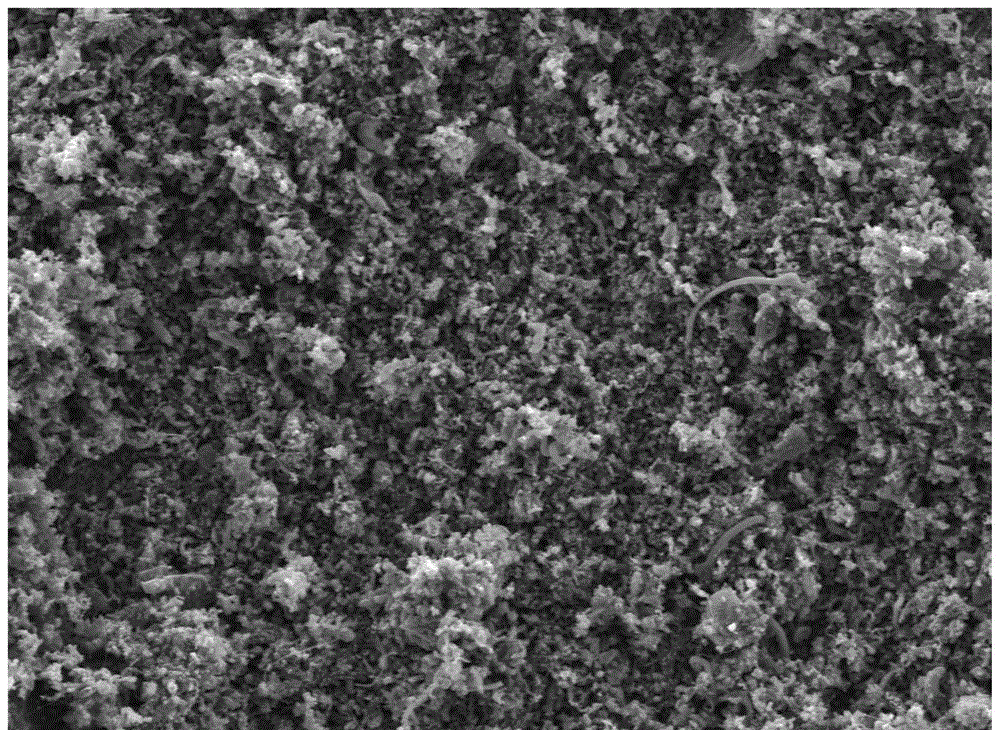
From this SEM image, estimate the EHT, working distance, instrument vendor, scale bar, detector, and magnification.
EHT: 5 kV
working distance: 9.6 mm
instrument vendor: JEOL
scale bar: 10000 nm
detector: LED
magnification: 2 K X